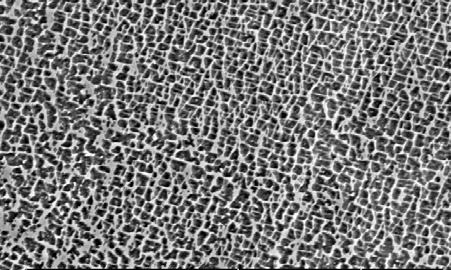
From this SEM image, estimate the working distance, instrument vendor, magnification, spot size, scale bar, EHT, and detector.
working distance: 7.4 mm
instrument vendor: FEI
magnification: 2.5 K X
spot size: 3.7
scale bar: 10000 nm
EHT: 20 kV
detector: BSE